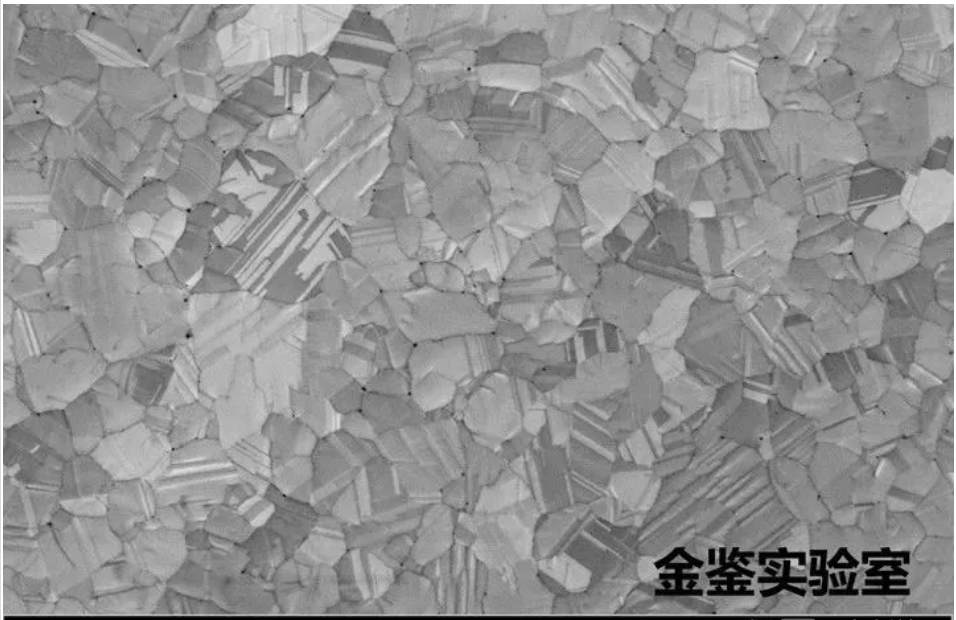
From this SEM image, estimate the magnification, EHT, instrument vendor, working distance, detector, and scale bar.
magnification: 10 K X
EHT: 5 kV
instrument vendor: Zeiss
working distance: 2.9 mm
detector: InLens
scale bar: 1000 nm